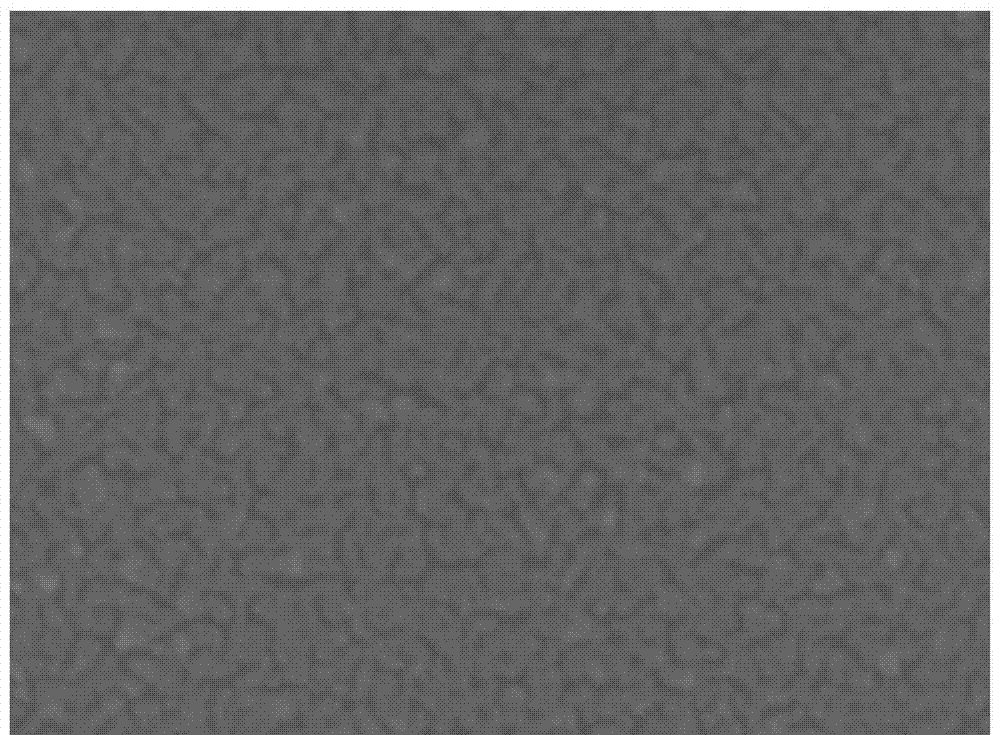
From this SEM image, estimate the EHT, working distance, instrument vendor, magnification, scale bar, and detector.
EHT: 10 kV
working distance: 3 mm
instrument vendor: JEOL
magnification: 100 K X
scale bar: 100 nm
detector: UED